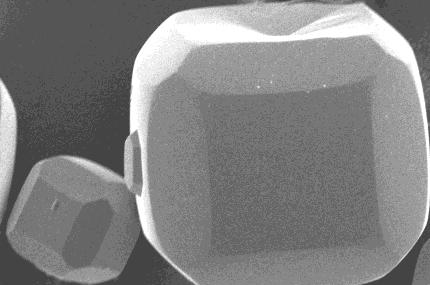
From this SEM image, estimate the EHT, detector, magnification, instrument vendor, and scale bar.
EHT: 10 kV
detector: SEI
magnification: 0.55 K X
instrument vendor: JEOL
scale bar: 20000 nm